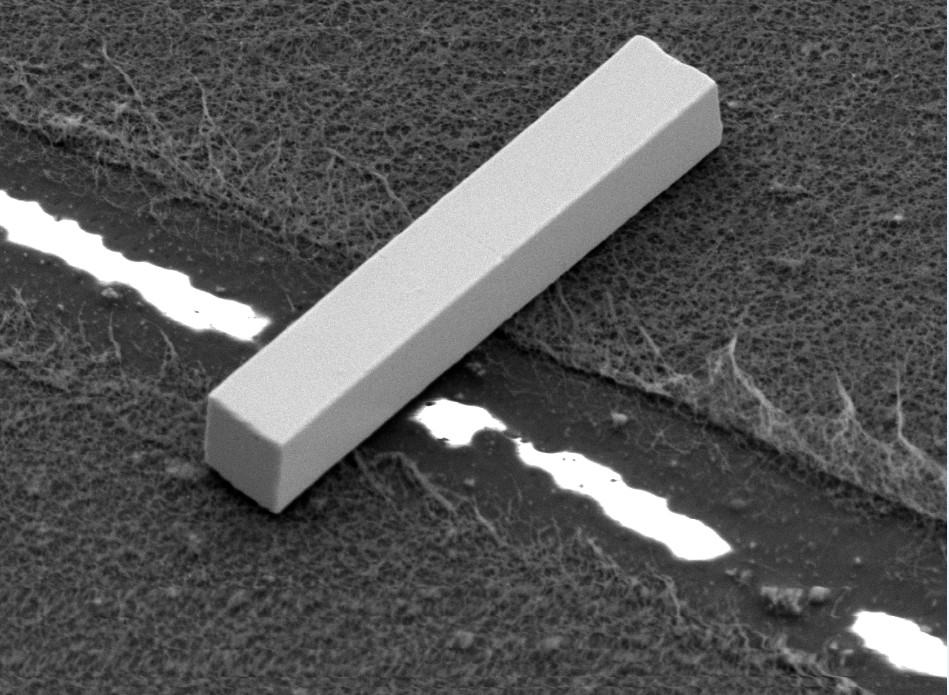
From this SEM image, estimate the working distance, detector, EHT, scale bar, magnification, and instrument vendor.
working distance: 14.9 mm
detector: SEI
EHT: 3 kV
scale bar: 1000 nm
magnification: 6 K X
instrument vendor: JEOL